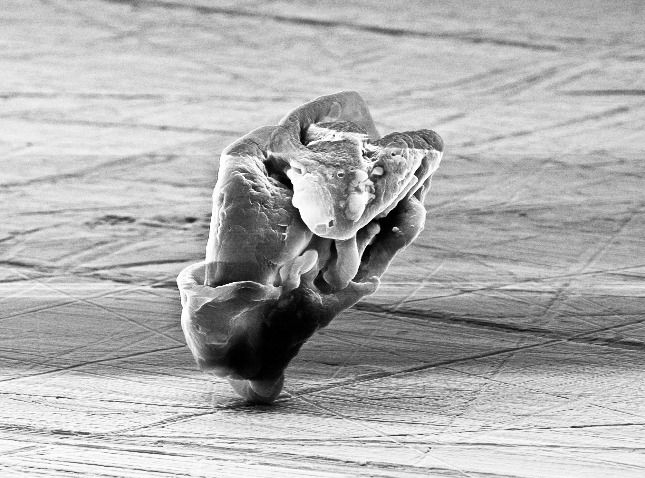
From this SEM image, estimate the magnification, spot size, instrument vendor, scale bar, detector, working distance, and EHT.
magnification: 4.337 K X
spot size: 3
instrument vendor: FEI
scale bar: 5000 nm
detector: SE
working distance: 16.3 mm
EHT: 10 kV